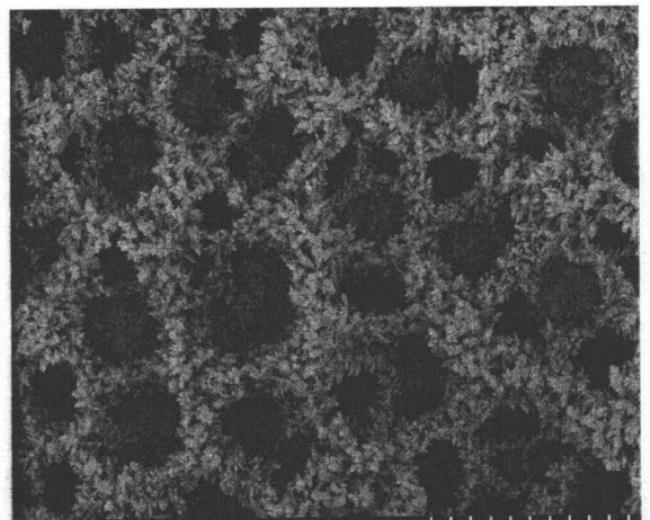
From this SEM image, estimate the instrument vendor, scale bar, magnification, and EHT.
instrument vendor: Hitachi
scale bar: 100000 nm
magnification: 0.4 K X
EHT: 5 kV